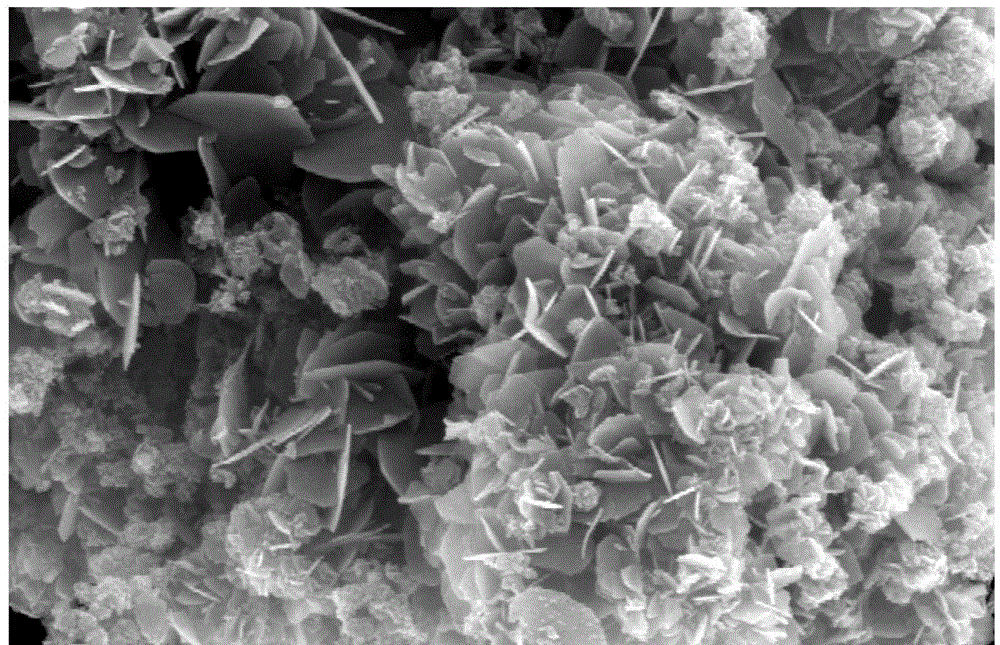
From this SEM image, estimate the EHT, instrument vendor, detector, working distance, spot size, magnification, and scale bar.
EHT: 15 kV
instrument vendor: FEI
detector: TLD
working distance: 6 mm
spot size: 5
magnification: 20 K X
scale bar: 1000 nm